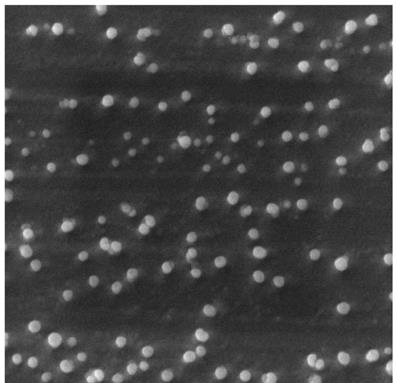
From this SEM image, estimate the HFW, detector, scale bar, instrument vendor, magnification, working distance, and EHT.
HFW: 7.22 µm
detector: SE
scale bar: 2000 nm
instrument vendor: Tescan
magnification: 30 K X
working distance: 10.18 mm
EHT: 20 kV